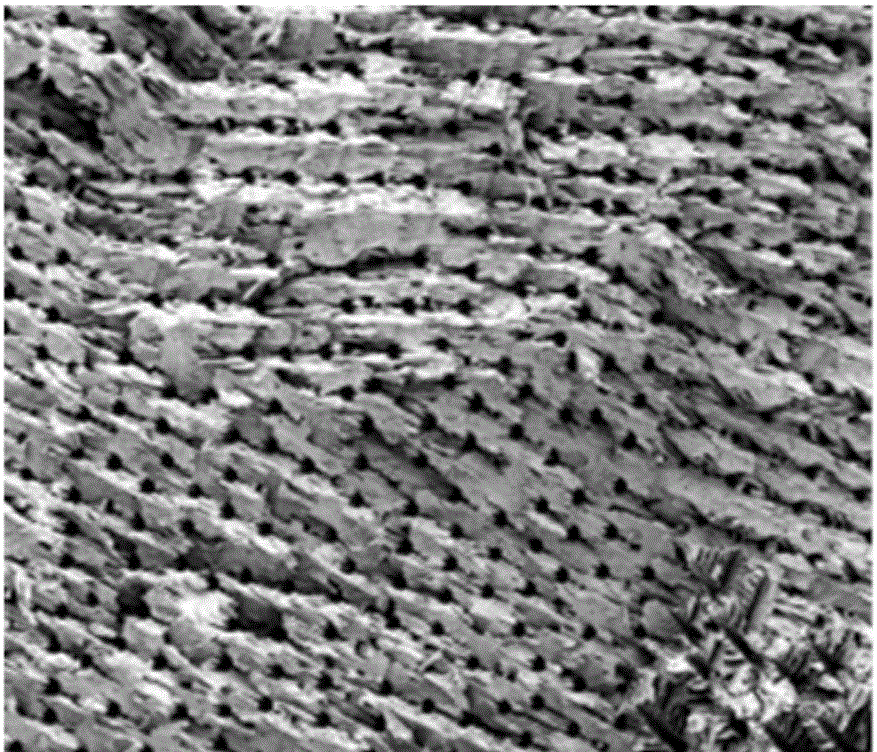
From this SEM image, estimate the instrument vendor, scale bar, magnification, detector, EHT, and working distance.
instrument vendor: FEI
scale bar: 500000 nm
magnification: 0.2 K X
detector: SE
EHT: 5 kV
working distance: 12.1 mm